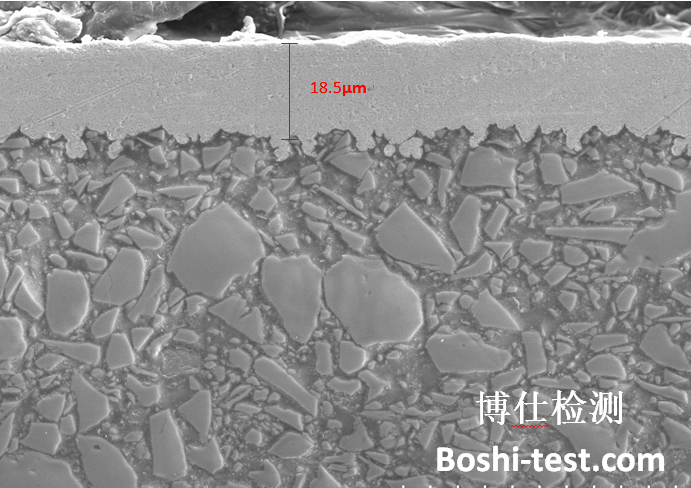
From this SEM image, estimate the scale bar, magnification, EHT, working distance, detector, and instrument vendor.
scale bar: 50000 nm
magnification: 1 K X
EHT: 10 kV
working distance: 6.8 mm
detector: SE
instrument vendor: Hitachi